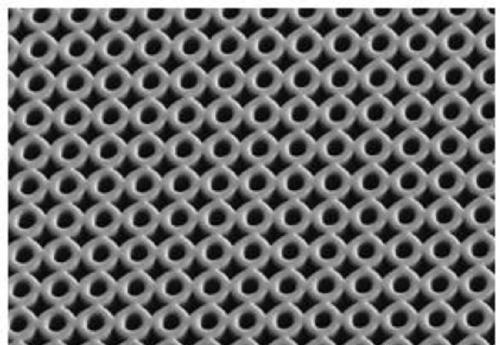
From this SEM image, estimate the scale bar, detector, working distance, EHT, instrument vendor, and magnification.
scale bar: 100000 nm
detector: SE(L)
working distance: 10 mm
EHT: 1 kV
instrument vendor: Hitachi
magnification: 0.5 K X